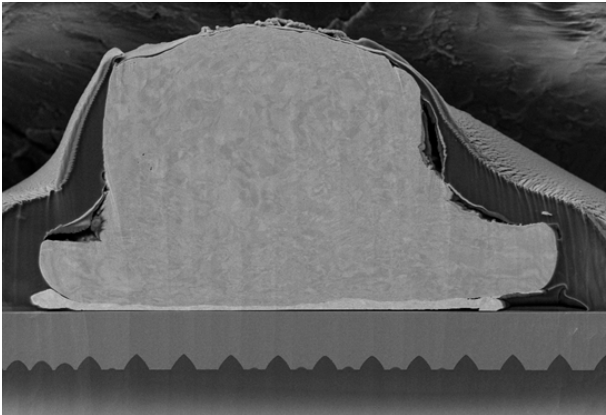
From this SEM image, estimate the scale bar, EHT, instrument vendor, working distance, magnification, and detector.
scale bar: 2000 nm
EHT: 5 kV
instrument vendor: Zeiss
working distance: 5.2 mm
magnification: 1.7 K X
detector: SE2